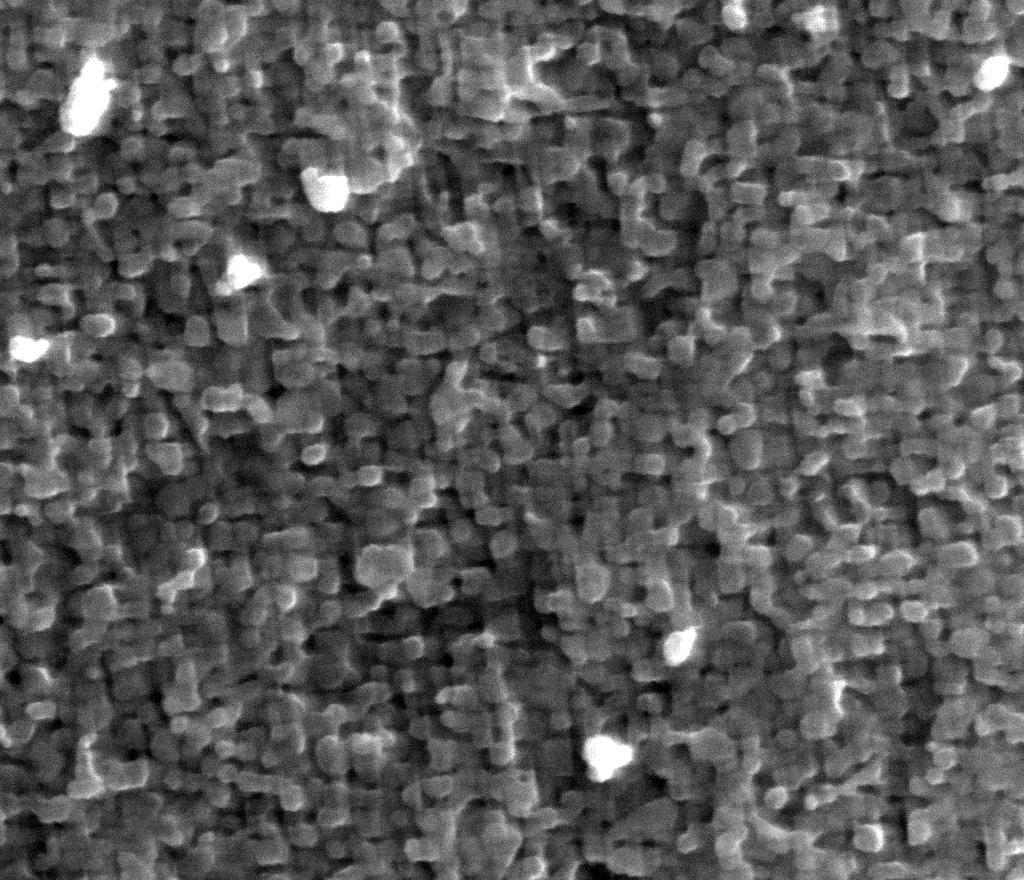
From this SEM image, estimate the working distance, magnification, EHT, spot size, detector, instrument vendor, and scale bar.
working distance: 10.2 mm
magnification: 100 K X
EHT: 20 kV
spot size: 4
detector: ETD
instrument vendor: FEI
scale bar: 500 nm